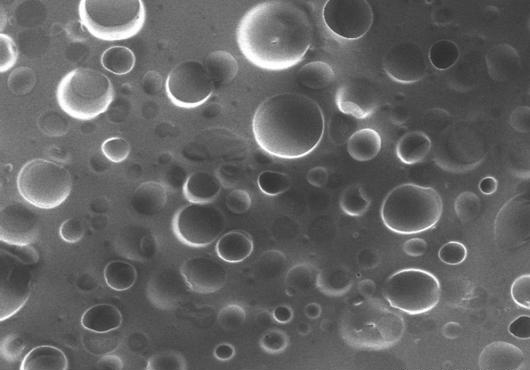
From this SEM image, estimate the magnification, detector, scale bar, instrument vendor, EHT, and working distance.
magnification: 0.6 K X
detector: SE(U)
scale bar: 50000 nm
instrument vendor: Hitachi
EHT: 10 kV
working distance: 5.7 mm